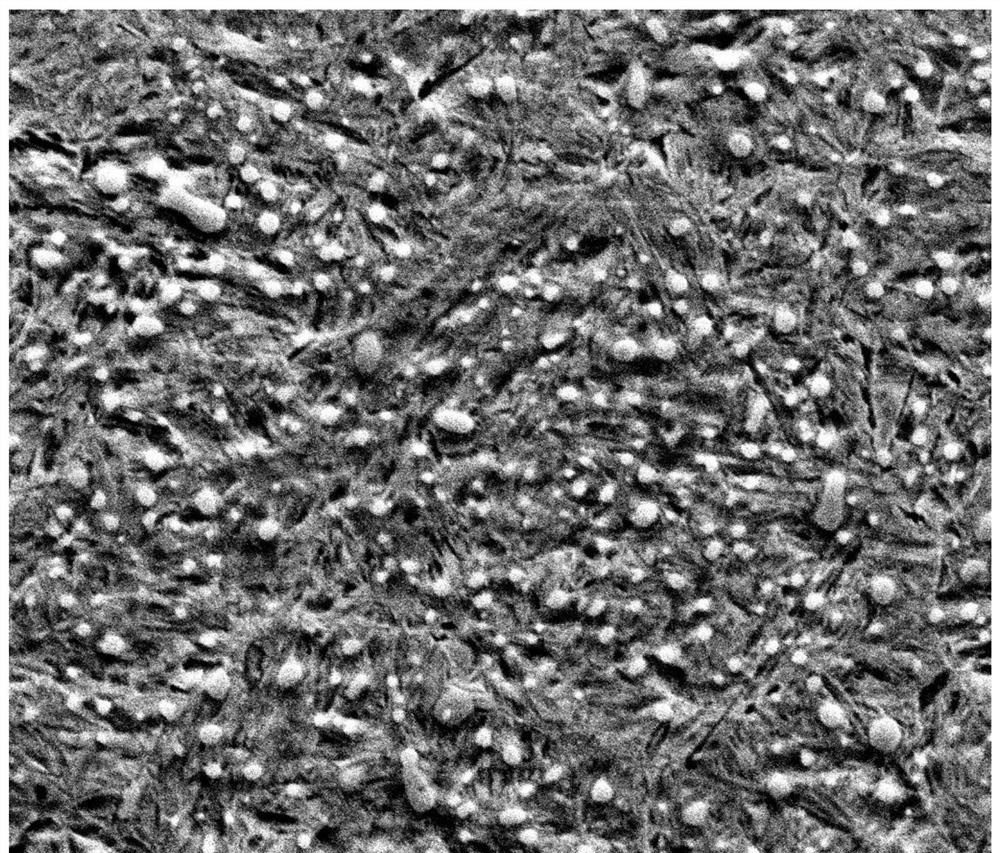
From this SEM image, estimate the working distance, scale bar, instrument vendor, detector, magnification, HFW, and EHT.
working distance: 22.2 mm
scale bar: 10000 nm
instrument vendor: FEI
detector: ETD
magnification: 5 K X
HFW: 29.8 µm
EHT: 20 kV